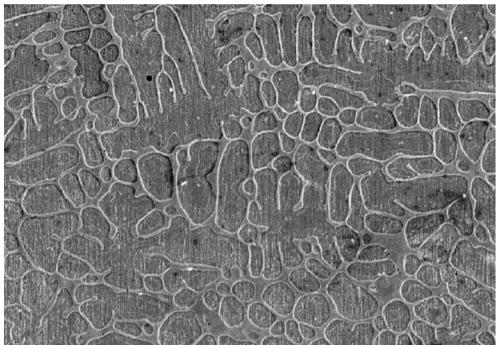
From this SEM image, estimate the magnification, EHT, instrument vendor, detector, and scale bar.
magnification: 1.4 K X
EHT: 10 kV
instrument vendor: Hitachi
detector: SE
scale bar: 40000 nm